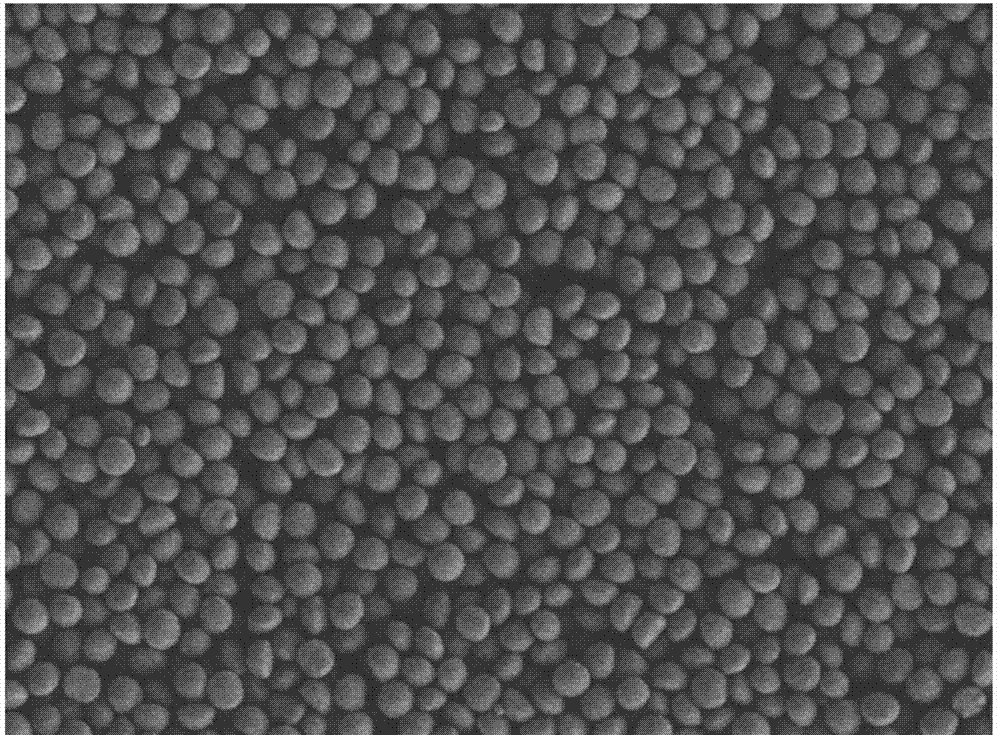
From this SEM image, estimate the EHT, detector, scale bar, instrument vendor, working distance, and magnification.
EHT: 5 kV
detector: LEI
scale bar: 10000 nm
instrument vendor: JEOL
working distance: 6.8 mm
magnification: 2 K X